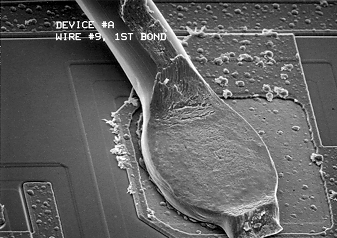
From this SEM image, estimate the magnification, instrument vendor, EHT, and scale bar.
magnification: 0.6 K X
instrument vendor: other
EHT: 10 kV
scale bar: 10000 nm